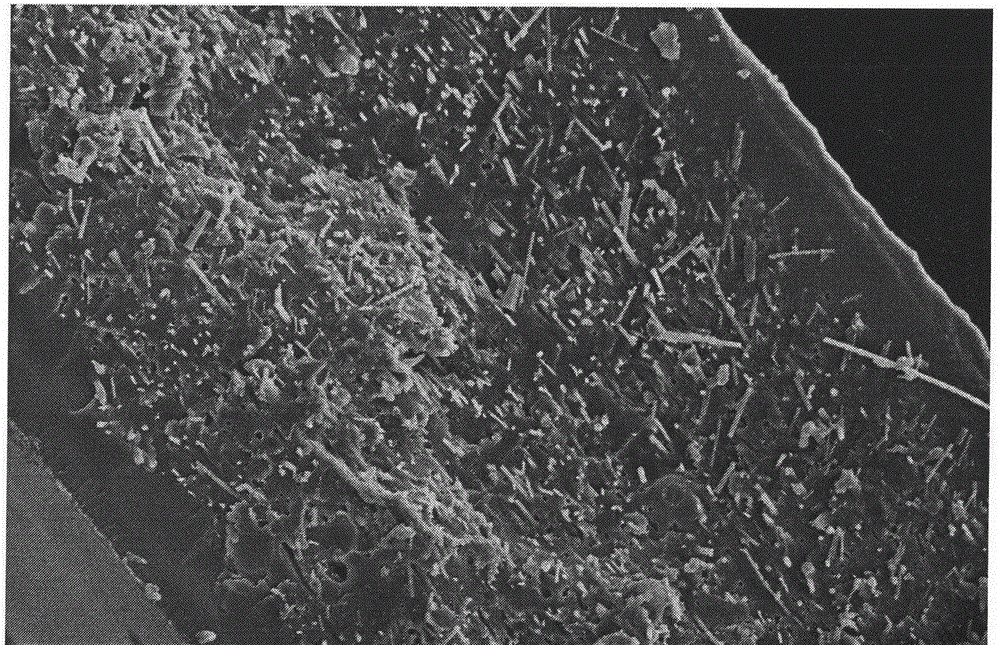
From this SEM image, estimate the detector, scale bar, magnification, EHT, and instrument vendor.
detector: SE(M)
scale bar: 50000 nm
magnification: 1 K X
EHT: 10 kV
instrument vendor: Hitachi